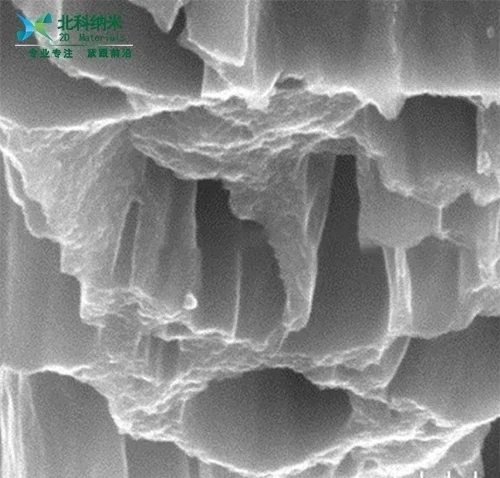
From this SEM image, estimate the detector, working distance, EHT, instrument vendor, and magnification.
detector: SE(U)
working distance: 8.6 mm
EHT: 5 kV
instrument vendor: Hitachi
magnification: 50 K X